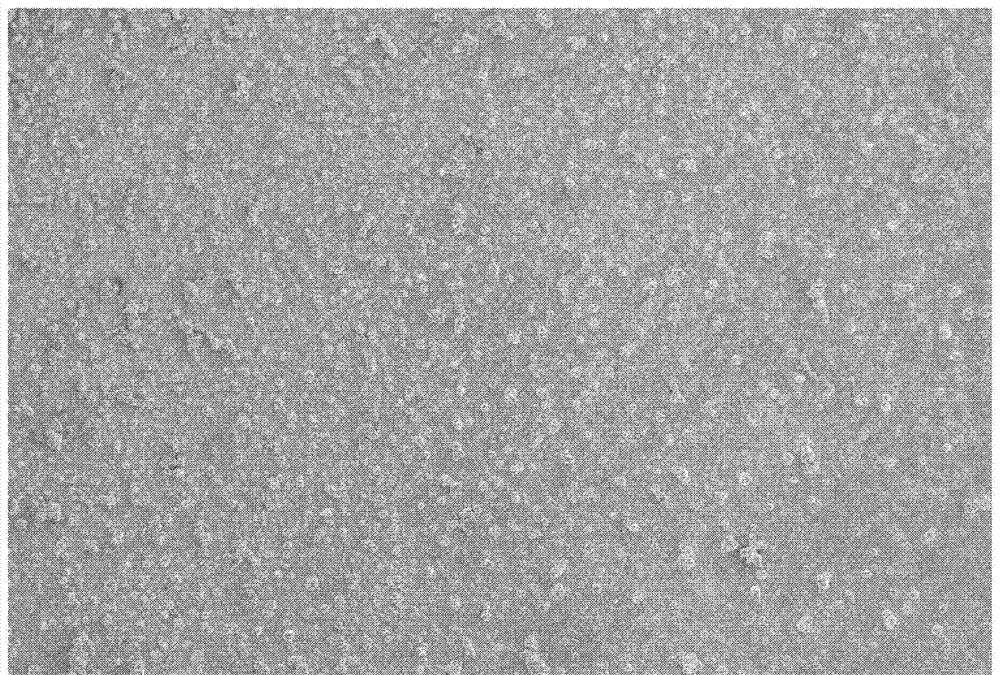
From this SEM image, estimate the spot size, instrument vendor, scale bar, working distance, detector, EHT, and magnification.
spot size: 30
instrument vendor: JEOL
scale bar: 500000 nm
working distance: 20 mm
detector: SEI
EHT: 10 kV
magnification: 0.03 K X